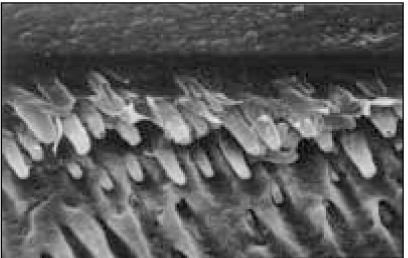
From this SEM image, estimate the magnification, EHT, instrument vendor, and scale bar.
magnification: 2 K X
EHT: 20 kV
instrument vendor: Hitachi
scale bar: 20000 nm